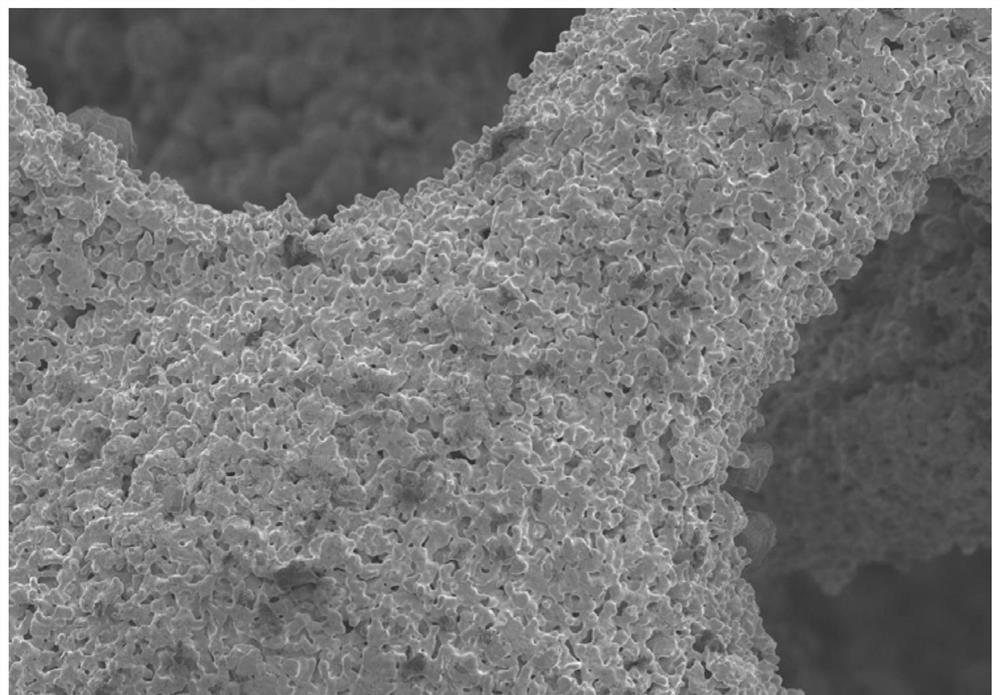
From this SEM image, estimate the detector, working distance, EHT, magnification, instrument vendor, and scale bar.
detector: SE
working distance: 11.9 mm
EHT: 5 kV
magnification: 0.1 K X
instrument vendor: Hitachi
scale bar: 500000 nm